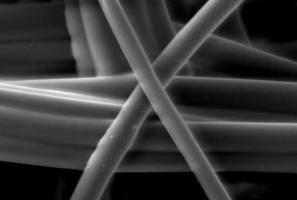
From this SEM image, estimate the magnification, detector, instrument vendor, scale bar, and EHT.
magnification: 2 K X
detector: SEI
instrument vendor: JEOL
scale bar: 10000 nm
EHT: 10 kV